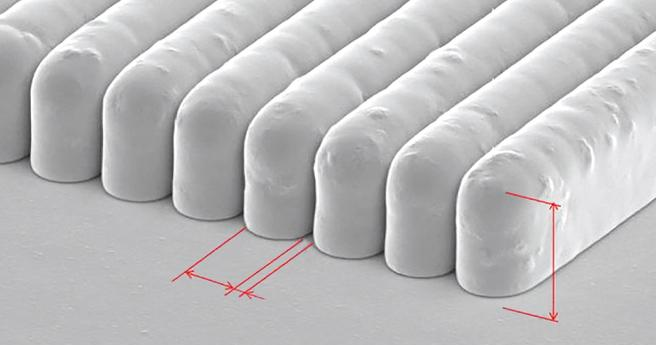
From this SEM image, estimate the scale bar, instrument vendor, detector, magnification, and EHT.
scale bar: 50000 nm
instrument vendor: JEOL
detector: SEI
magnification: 0.5 K X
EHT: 15 kV